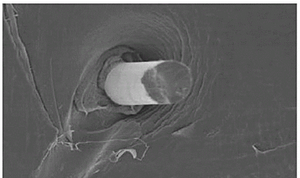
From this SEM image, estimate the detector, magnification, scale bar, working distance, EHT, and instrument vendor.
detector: SE(U)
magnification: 2 K X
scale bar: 20000 nm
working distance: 6.5 mm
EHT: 5 kV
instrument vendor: Hitachi